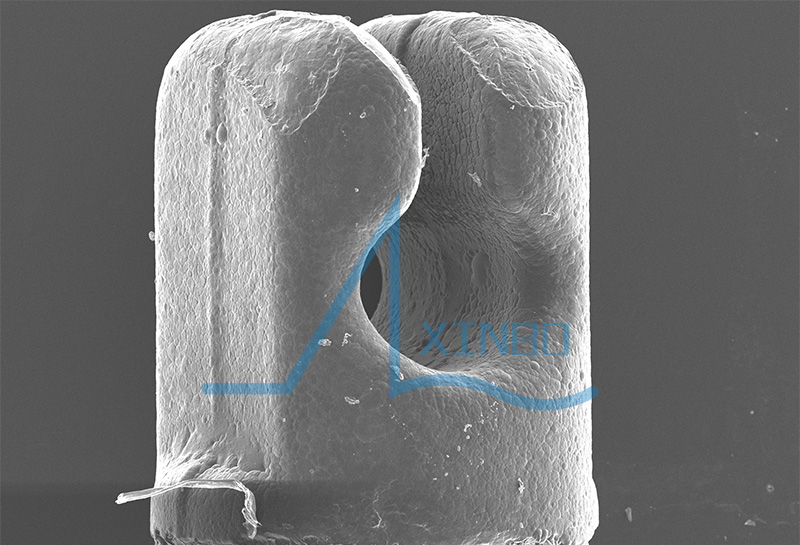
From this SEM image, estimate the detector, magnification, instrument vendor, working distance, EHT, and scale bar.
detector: SED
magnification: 0.07 K X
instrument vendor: JEOL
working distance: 20 mm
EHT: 10 kV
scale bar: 200000 nm